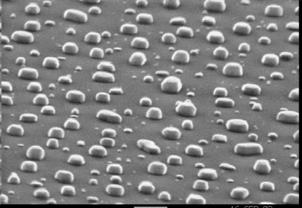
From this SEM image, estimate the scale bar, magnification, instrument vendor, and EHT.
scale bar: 1000 nm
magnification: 13.6 K X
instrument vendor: JEOL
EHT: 30 kV